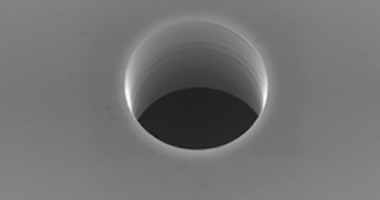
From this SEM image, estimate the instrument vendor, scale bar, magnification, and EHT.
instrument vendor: JEOL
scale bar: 5000 nm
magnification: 3.5 K X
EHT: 25 kV